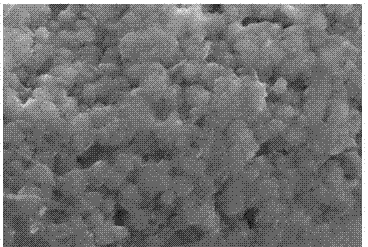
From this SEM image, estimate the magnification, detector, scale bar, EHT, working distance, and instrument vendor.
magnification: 20 K X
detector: SE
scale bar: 2000 nm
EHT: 15 kV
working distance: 5.7 mm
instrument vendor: Hitachi